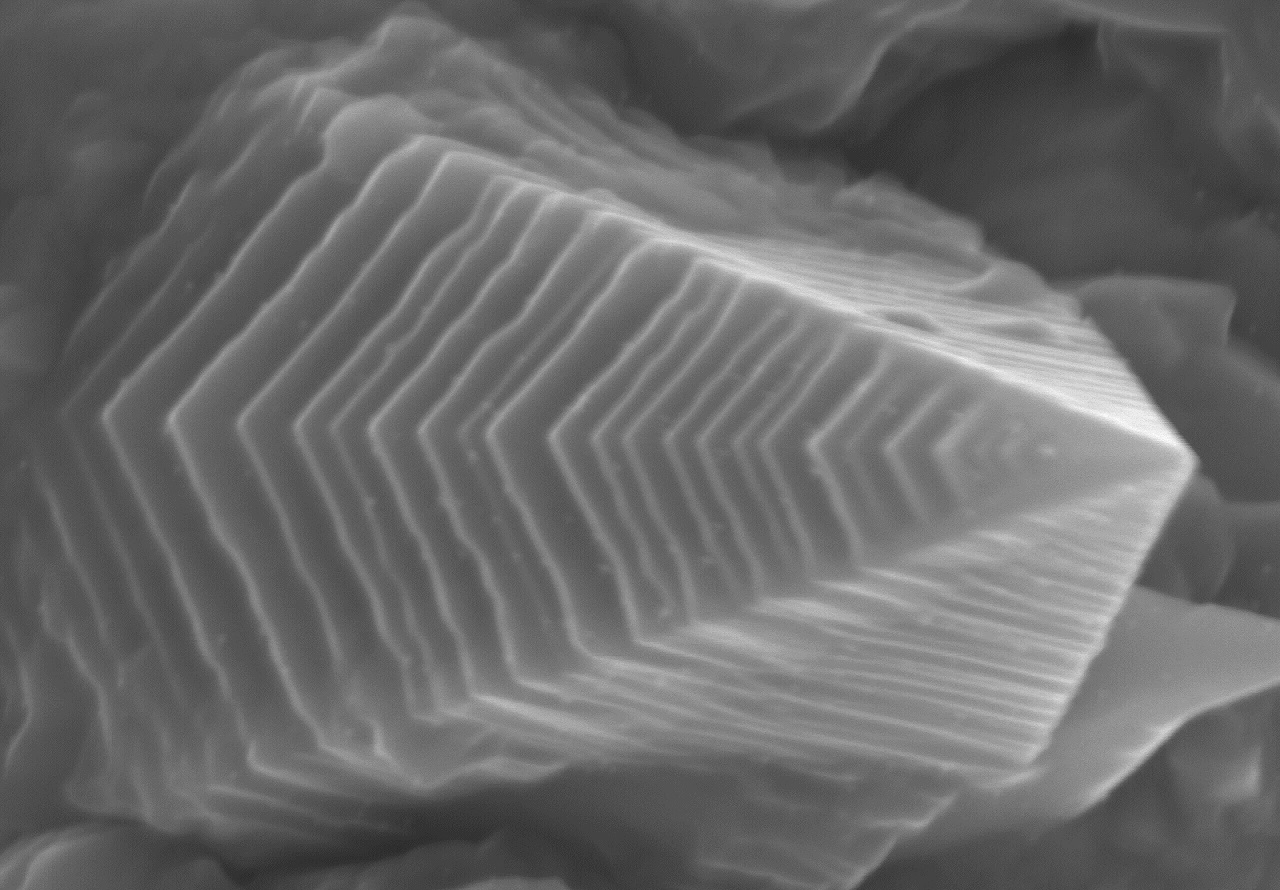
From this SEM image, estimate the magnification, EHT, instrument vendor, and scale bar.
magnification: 70 K X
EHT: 5 kV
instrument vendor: Hitachi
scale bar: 500 nm